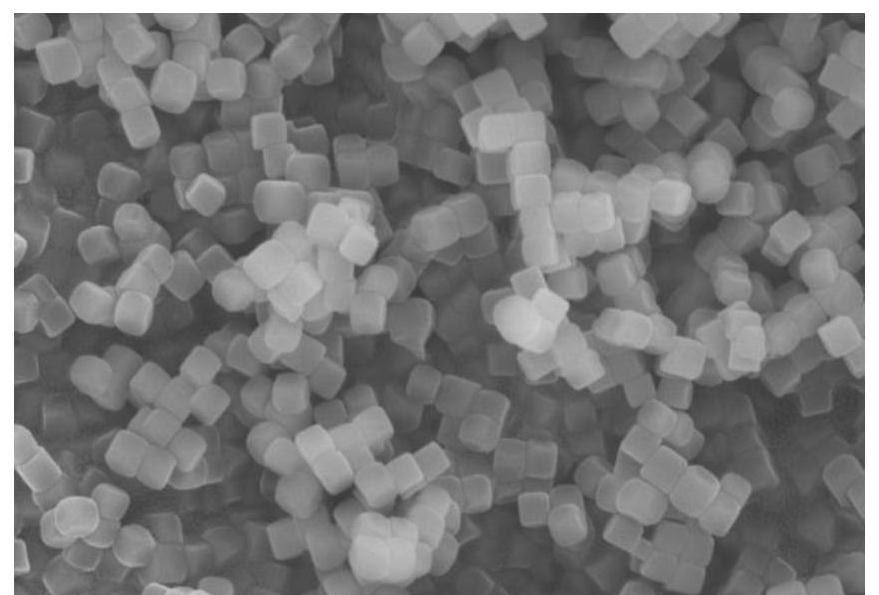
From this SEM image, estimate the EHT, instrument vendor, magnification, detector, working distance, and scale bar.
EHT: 11 kV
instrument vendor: Hitachi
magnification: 30 K X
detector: SE(UL)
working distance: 13.3 mm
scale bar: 1000 nm